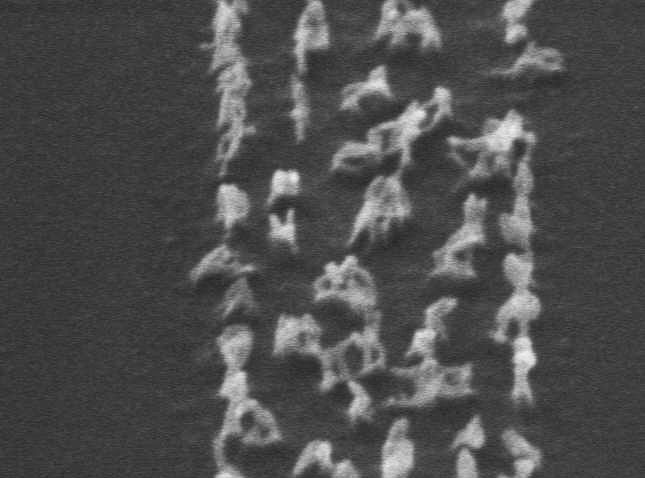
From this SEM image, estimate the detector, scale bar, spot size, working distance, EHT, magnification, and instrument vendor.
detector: SE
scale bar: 200 nm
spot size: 3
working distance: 11.4 mm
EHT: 10 kV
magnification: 74.927 K X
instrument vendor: FEI